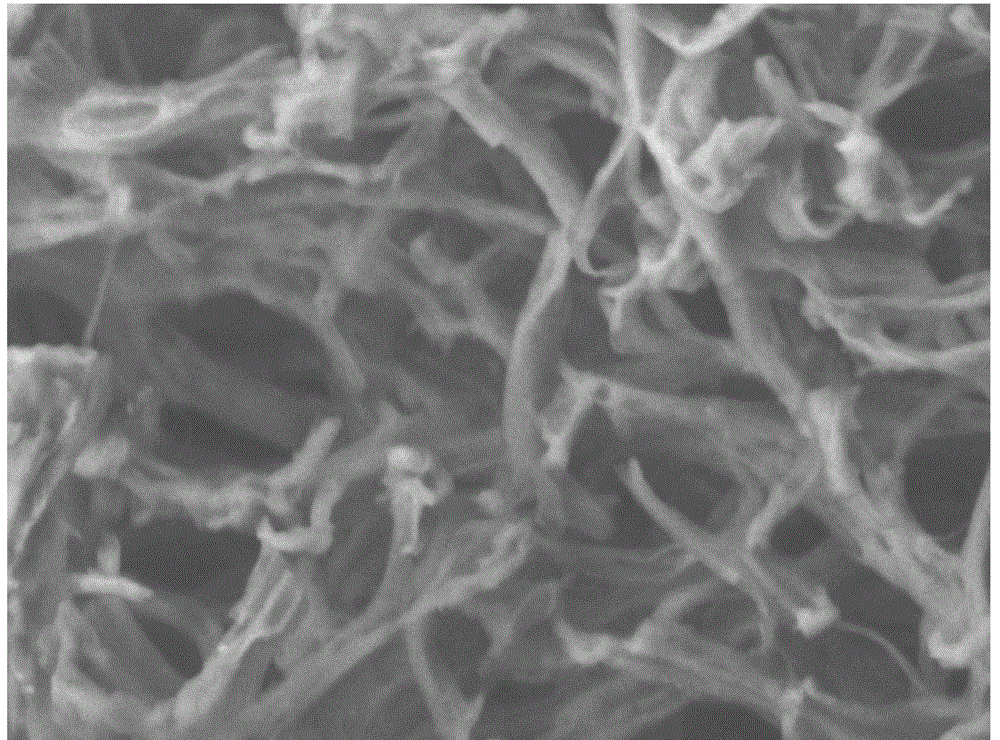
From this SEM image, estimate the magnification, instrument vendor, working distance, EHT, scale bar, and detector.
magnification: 10 K X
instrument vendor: JEOL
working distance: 8.1 mm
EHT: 3 kV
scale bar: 1000 nm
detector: LEI